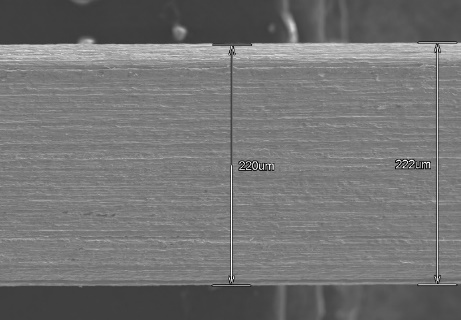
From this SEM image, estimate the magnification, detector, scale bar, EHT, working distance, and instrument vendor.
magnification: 0.3 K X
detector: SE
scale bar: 100000 nm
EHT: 15 kV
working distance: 12 mm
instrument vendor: Hitachi